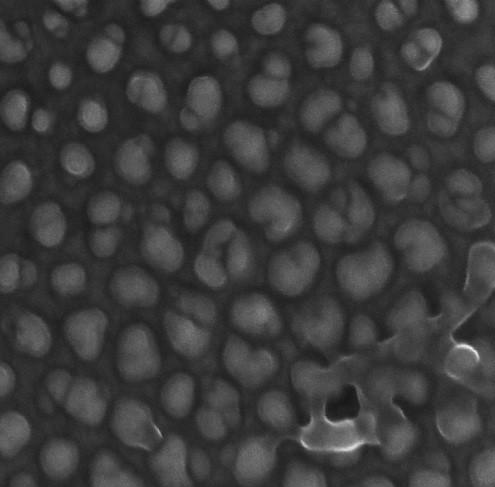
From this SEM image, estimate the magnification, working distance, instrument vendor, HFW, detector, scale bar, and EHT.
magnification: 250 K X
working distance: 2.987 mm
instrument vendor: Tescan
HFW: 0.867 µm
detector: InBeam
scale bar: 200 nm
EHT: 15 kV